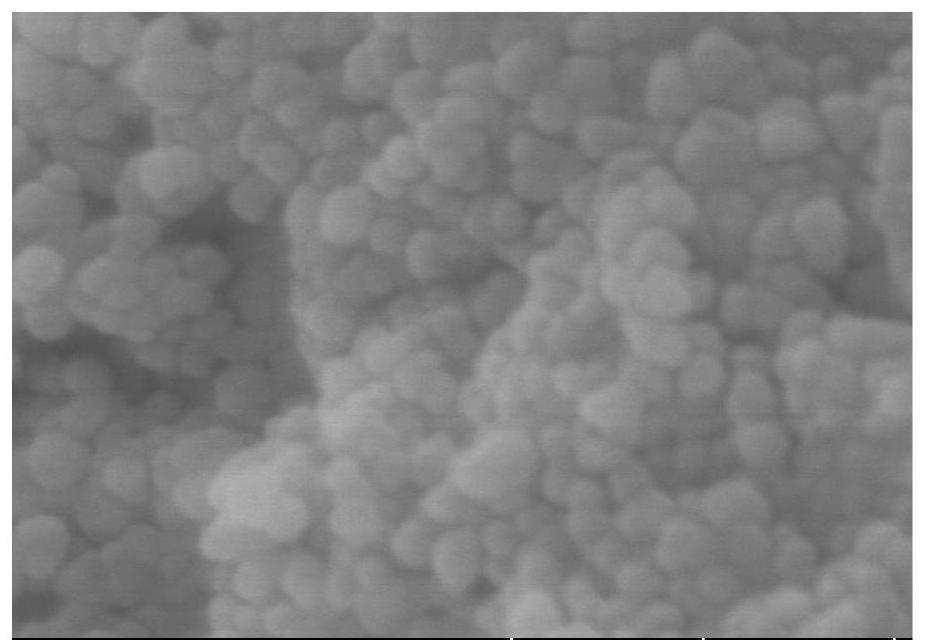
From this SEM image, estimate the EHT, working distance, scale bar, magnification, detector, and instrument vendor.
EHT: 5 kV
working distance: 7.8 mm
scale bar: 300 nm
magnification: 180 K X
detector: SE(UL)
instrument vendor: Hitachi